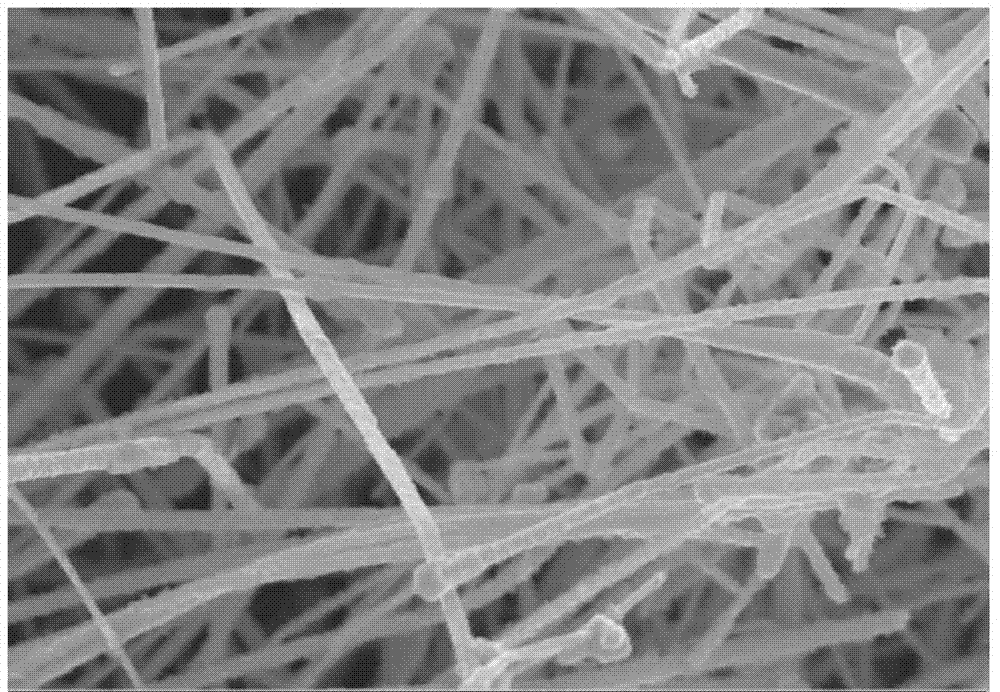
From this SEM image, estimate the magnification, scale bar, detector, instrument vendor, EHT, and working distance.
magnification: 19.51 K X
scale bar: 200 nm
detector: InLens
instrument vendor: Zeiss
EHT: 8 kV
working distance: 3 mm